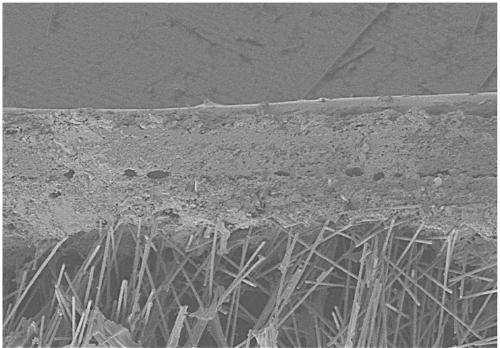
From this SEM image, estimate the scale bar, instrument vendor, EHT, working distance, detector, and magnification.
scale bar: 500000 nm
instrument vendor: Hitachi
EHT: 5 kV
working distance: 13.8 mm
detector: SE(M)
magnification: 0.1 K X